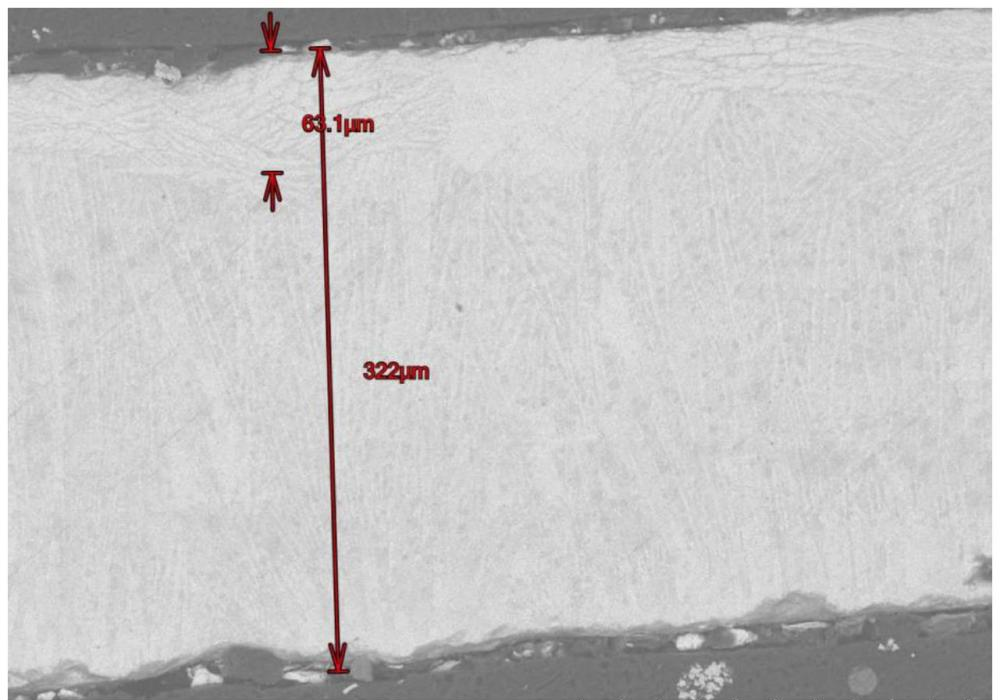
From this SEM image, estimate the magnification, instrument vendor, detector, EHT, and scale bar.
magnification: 0.25 K X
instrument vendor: Hitachi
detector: BSE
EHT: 15 kV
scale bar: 200000 nm